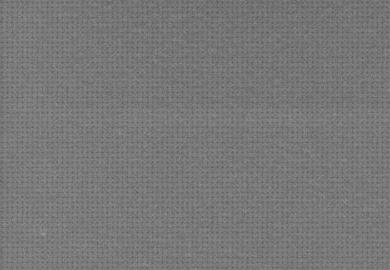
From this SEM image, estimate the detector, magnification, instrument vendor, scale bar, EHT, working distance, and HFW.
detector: SE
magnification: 7 K X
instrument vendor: Tescan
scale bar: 5000 nm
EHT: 1.5 kV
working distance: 9.25 mm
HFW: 29.7 µm